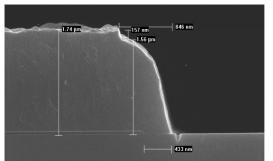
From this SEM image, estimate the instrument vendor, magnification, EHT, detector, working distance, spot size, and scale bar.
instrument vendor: FEI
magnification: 30 K X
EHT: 10 kV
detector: TLD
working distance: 5.1 mm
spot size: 3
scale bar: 1000 nm